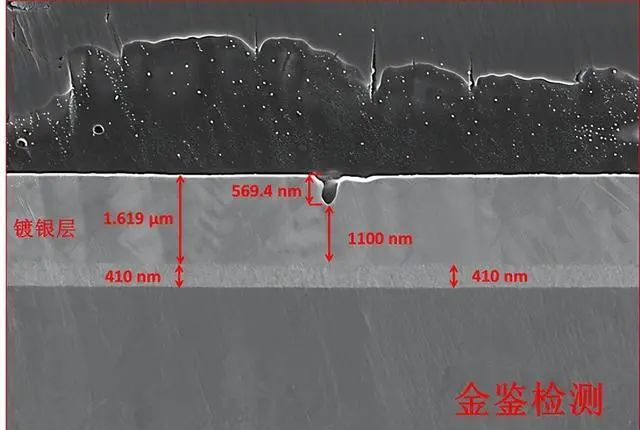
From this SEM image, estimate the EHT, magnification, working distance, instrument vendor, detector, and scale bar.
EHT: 5 kV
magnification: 10 K X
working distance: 4.3 mm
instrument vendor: Zeiss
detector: InLens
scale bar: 1000 nm